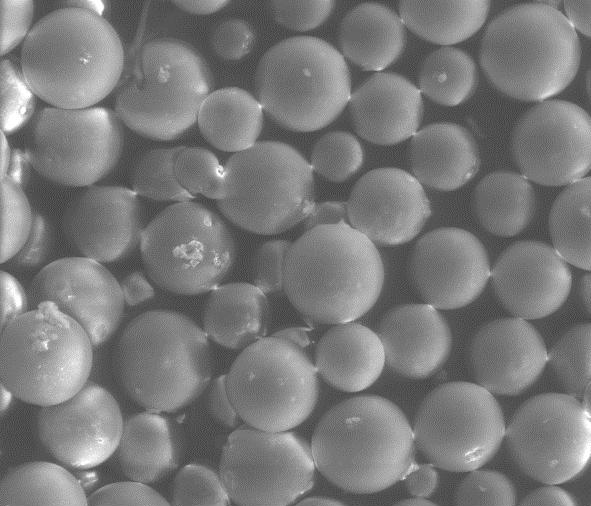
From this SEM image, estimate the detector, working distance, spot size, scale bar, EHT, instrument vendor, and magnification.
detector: LFD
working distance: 10.7 mm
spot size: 5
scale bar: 300000 nm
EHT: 25 kV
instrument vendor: FEI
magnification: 0.2 K X